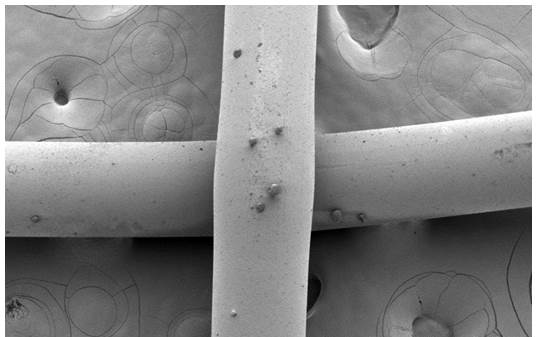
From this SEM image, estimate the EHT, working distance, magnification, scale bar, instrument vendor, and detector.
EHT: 1 kV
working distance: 11.1 mm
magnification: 0.1 K X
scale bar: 500000 nm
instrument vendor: Hitachi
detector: SE(M)